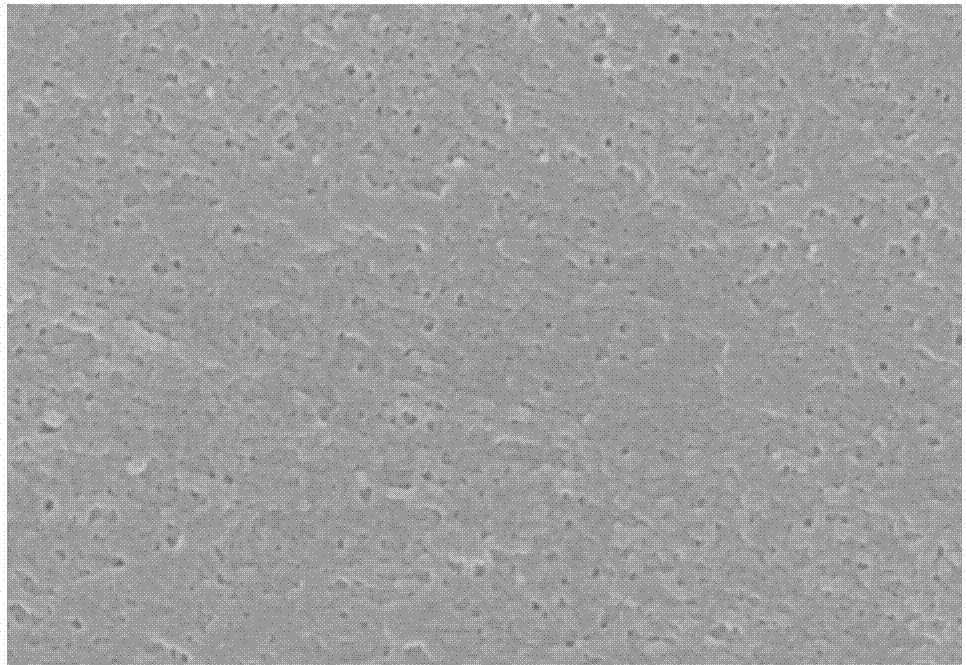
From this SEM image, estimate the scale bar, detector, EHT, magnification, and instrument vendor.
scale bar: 1000 nm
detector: SE(M)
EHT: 8 kV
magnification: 40 K X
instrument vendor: Hitachi